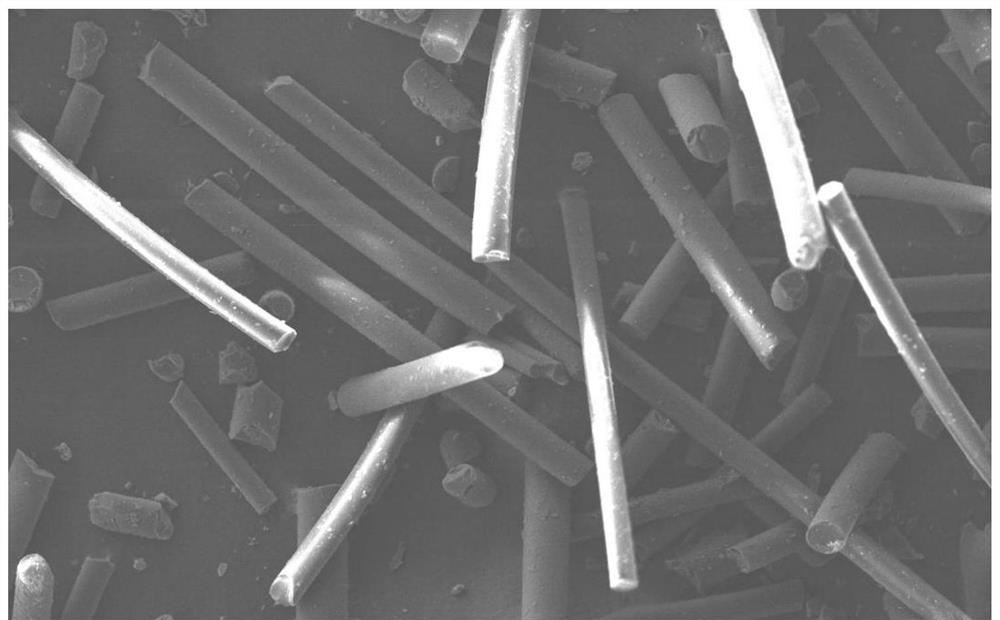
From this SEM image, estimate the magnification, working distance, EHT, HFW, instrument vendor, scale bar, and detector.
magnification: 1 K X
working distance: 4.846 mm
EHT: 10 kV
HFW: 518 µm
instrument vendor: Thermo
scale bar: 150000 nm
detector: SED